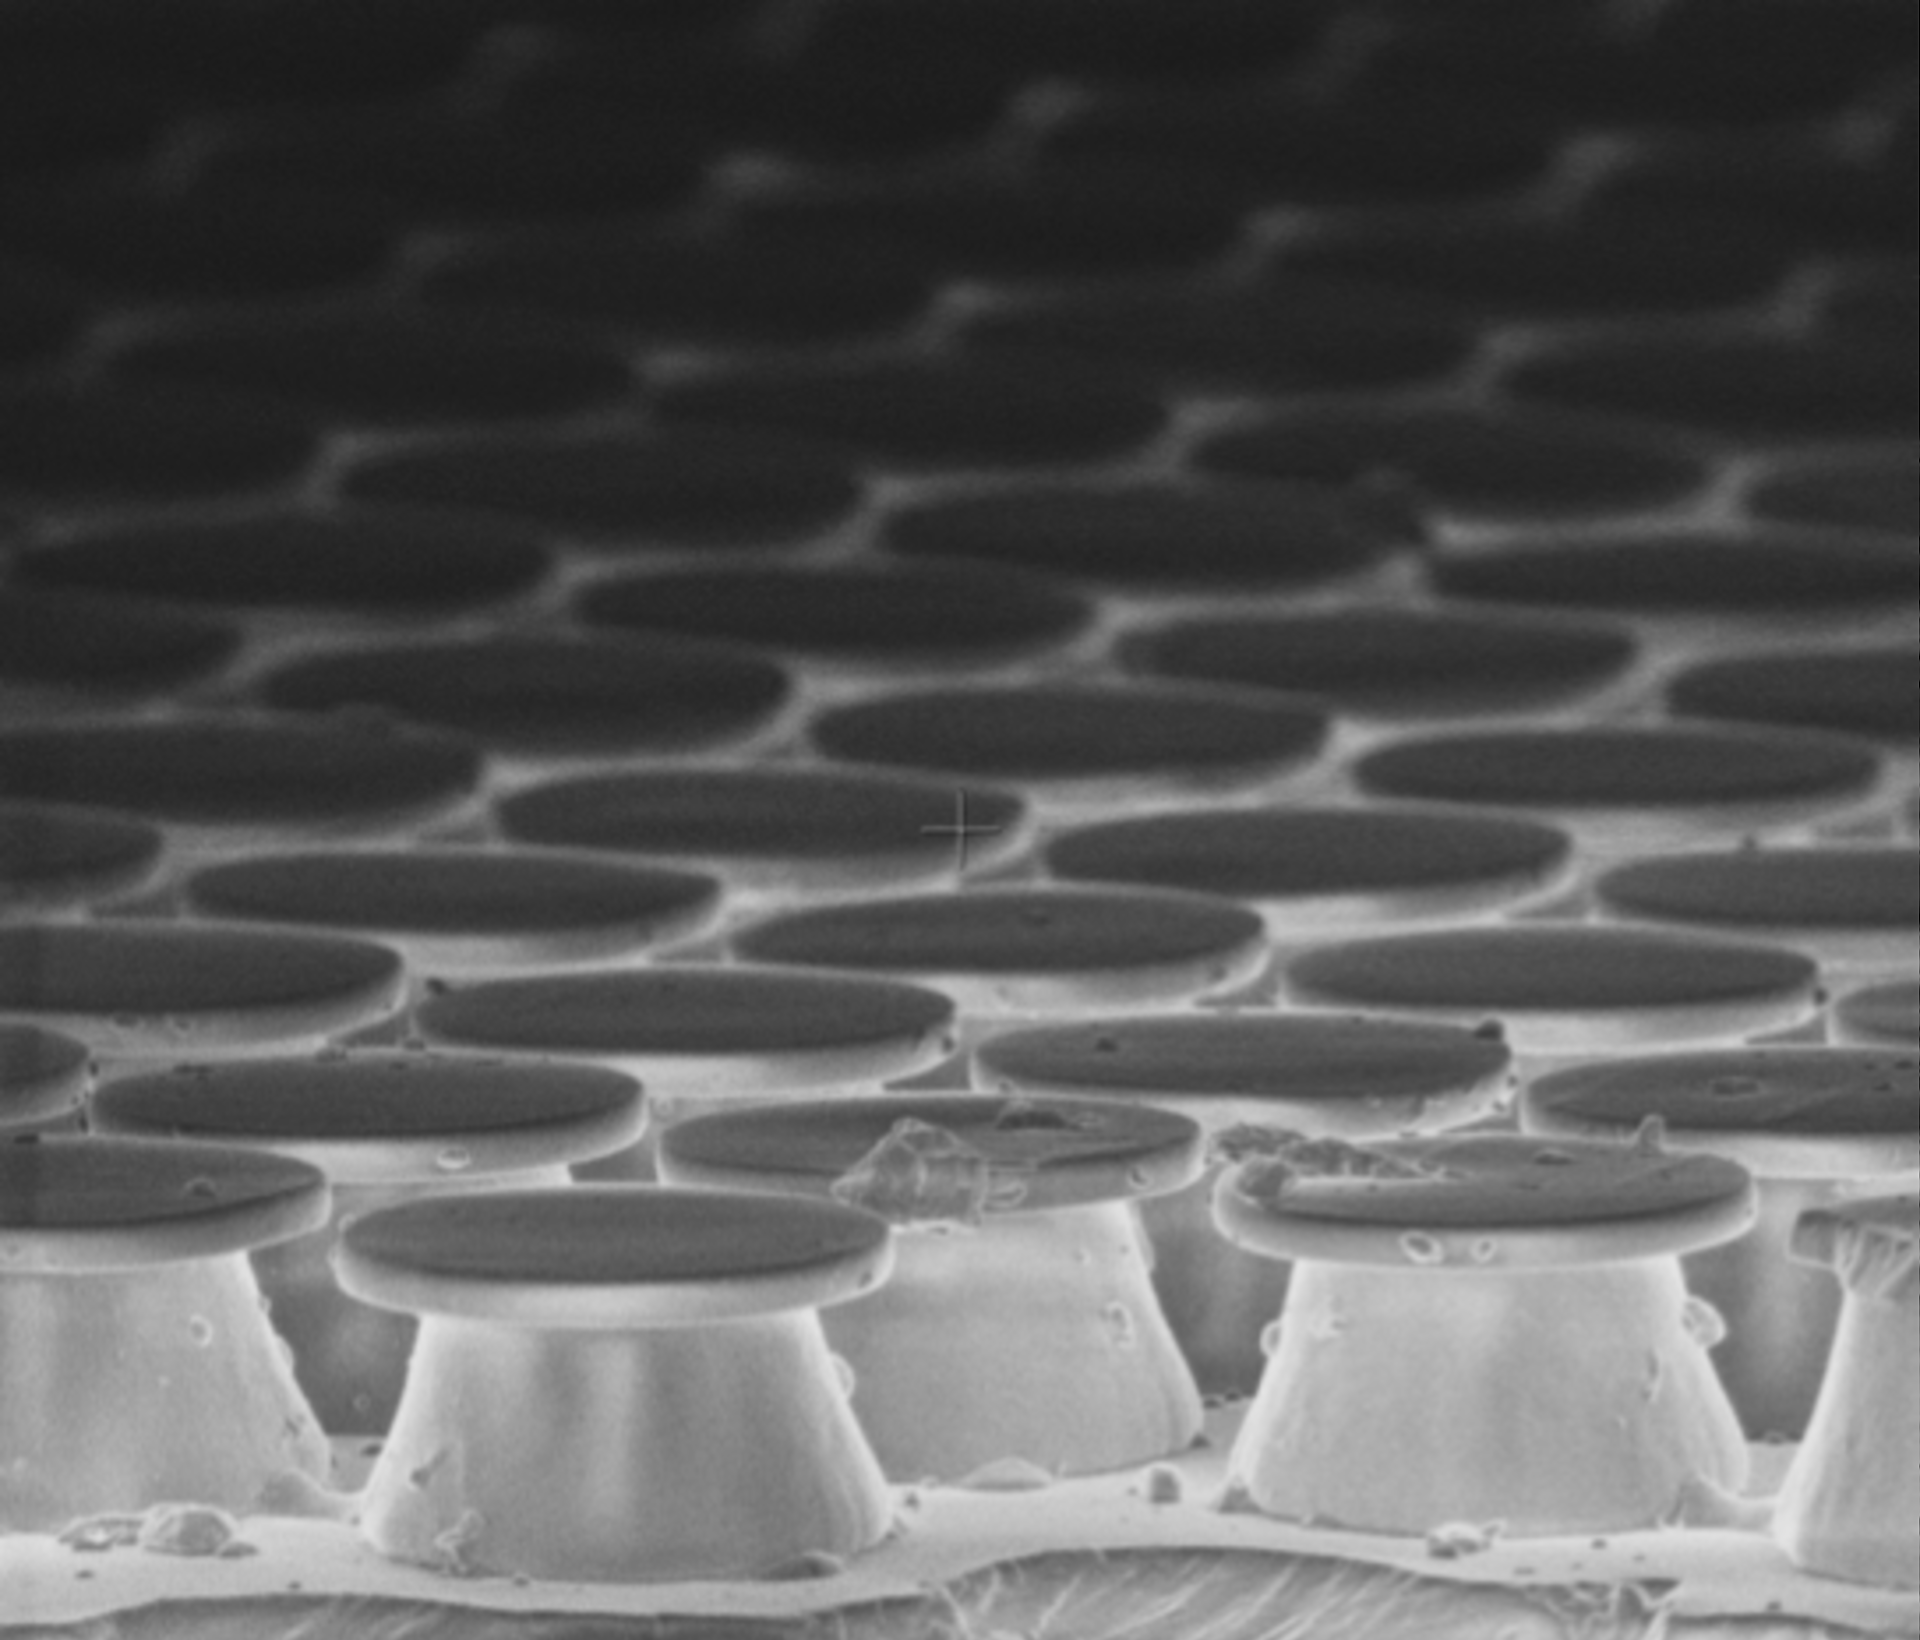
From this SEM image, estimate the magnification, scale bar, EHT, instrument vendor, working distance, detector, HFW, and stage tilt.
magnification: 5 K X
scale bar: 10000 nm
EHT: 2 kV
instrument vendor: FEI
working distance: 5.8 mm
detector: TLD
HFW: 59.7 µm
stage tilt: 10°